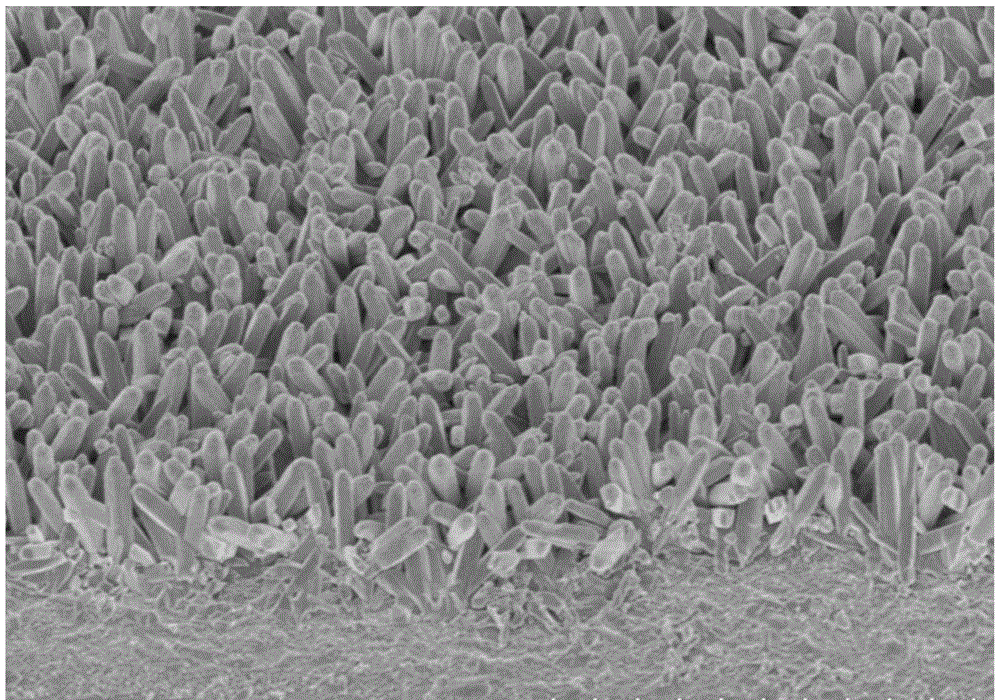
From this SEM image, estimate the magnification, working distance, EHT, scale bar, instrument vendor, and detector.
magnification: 18 K X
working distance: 9.1 mm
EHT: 1 kV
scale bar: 3000 nm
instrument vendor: Hitachi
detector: SE(U)